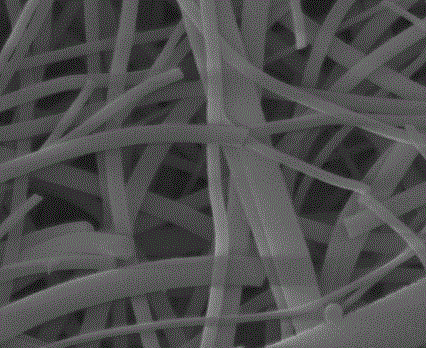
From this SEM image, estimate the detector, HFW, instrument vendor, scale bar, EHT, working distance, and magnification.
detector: ETD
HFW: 7.46 µm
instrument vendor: FEI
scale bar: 3000 nm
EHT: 10 kV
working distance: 12 mm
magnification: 40 K X